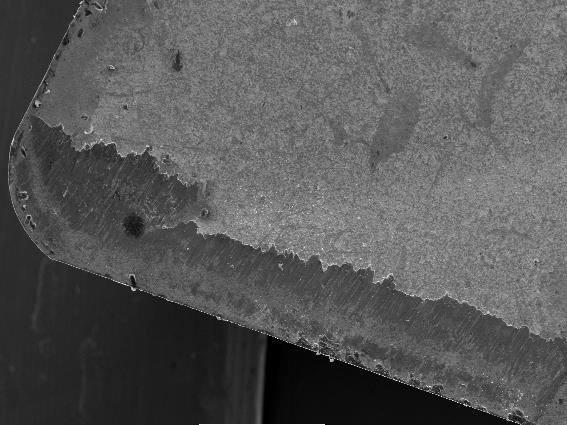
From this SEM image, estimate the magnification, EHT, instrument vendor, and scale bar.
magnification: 0.05 K X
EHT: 20 kV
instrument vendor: JEOL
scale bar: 500000 nm